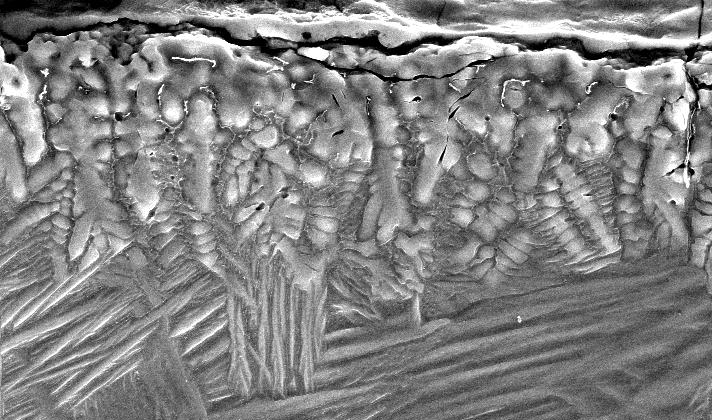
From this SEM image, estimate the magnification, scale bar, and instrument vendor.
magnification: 1 K X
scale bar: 20000 nm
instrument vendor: FEI